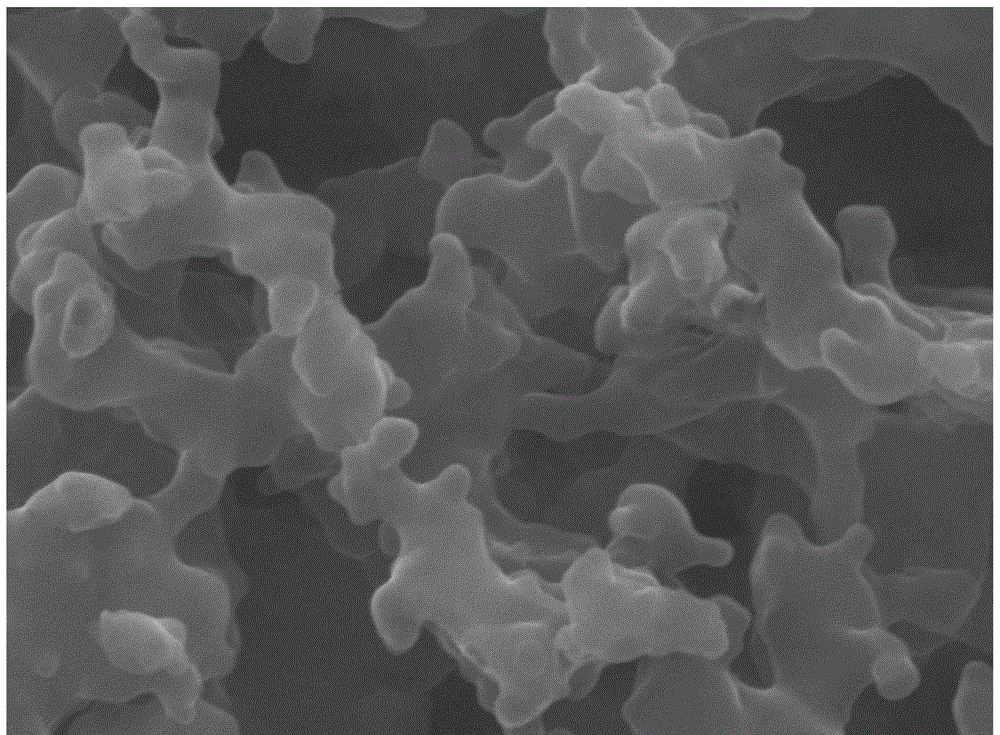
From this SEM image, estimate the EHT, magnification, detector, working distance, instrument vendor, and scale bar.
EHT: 20 kV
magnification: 50 K X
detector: LED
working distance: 11.4 mm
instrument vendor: JEOL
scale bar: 100 nm